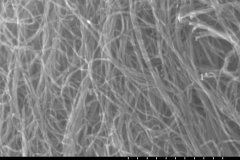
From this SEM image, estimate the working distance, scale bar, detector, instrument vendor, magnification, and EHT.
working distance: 8.4 mm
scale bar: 500 nm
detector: SE(M)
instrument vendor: Hitachi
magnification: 100 K X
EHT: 20 kV